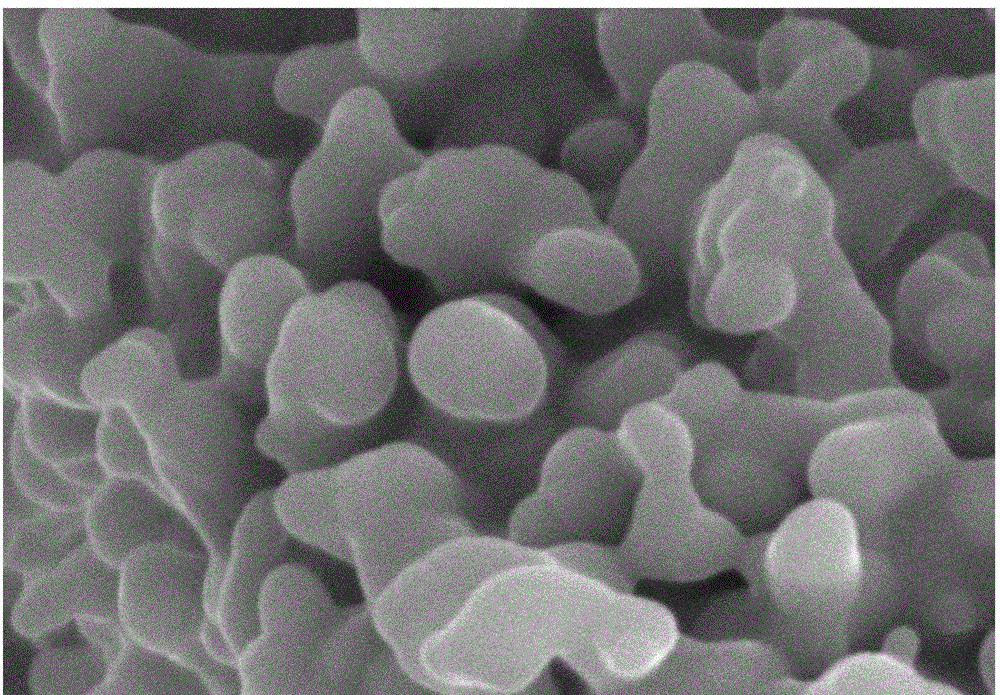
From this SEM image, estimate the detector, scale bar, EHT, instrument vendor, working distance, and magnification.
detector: SE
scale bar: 2000 nm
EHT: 30 kV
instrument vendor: Hitachi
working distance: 9.9 mm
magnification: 20 K X